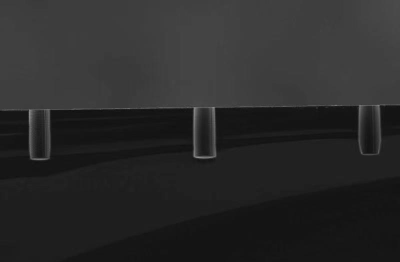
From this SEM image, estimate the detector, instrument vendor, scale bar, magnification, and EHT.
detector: SEI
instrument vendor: JEOL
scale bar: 100000 nm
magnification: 0.2 K X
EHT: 20 kV